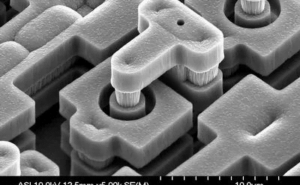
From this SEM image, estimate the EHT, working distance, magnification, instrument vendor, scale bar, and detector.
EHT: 10 kV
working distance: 12.5 mm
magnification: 5 K X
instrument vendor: Hitachi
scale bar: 10000 nm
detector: SE(M)